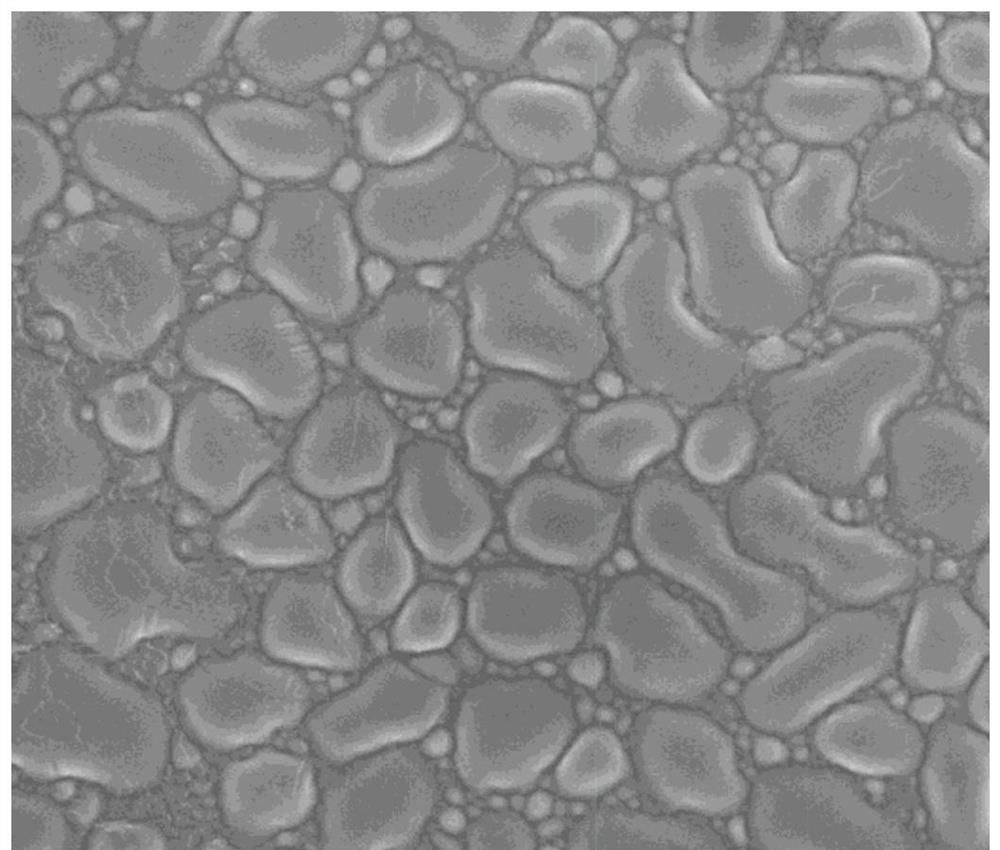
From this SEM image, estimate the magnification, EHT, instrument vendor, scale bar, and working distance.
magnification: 5 K X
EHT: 10 kV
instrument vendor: FEI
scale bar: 10000 nm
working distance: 5.5 mm